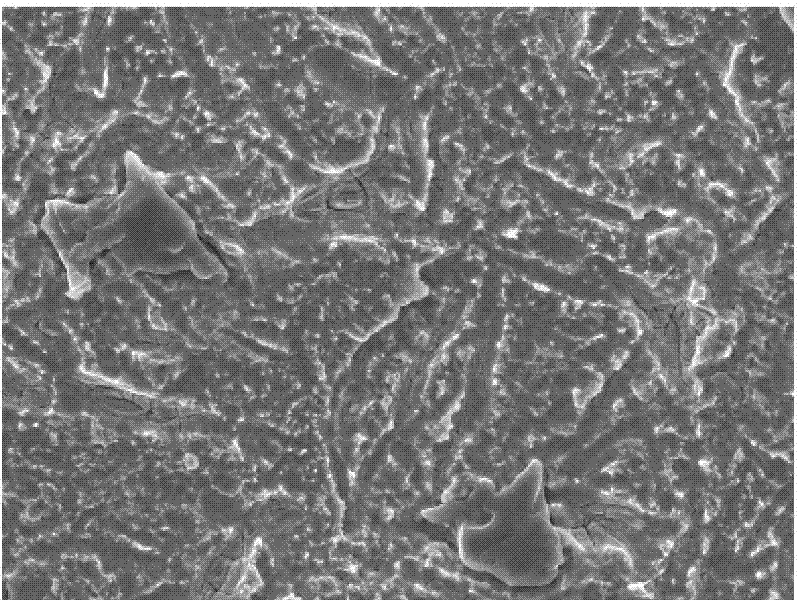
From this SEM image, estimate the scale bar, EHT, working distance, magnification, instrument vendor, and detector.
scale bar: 10000 nm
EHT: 20 kV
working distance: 8.1 mm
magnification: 0.75 K X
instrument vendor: JEOL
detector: SEI